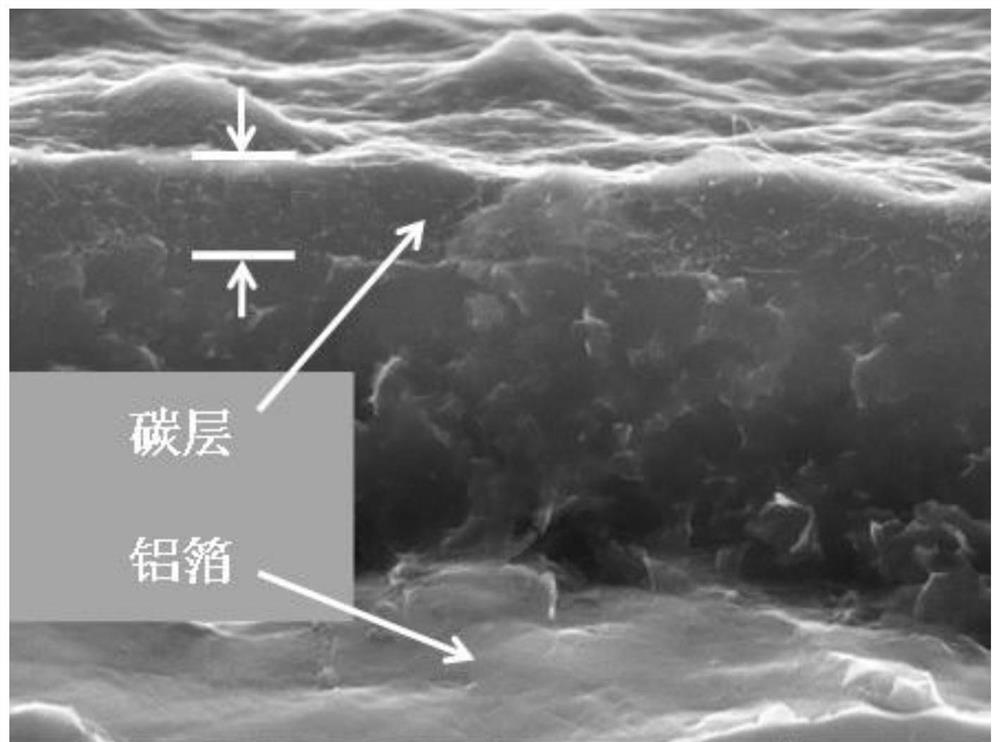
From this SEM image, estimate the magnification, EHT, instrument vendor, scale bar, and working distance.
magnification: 40 K X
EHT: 20 kV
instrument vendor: Tescan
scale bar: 2000 nm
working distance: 14.72 mm